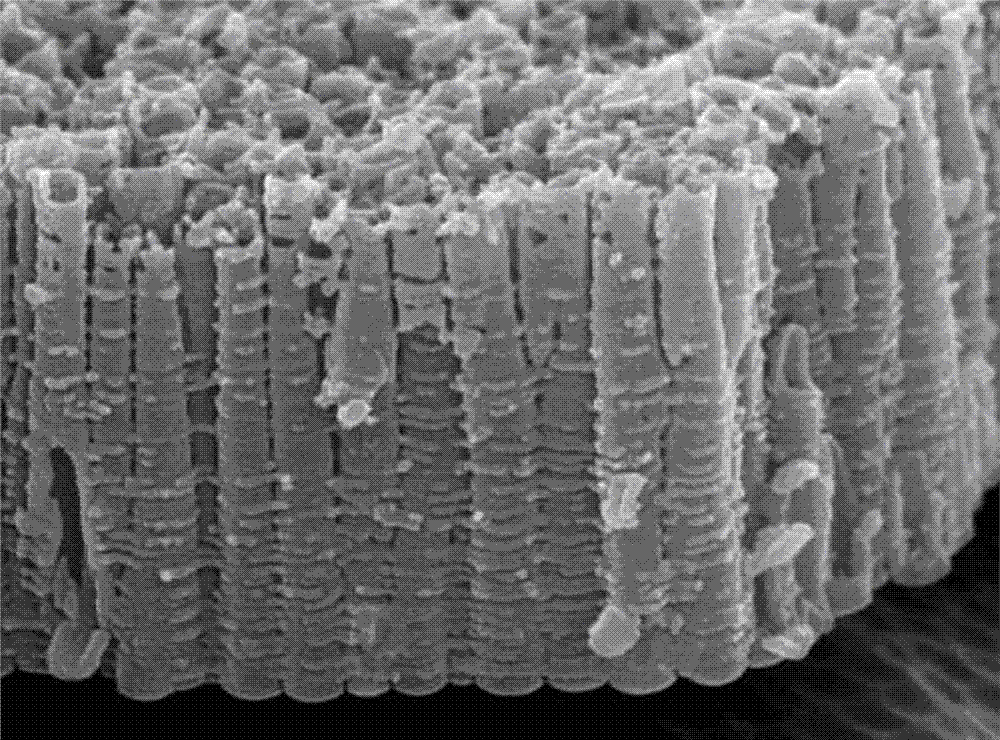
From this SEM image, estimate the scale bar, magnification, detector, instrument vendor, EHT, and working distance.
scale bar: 100 nm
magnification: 50 K X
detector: SEI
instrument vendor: JEOL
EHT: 15 kV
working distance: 4.8 mm